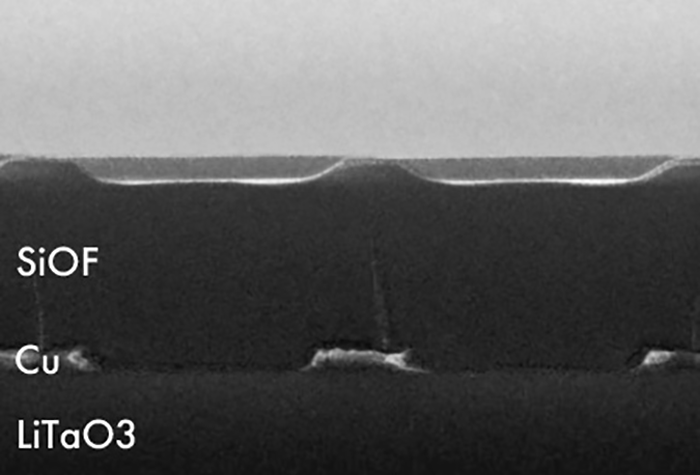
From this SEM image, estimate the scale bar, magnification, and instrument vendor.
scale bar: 1000 nm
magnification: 15 K X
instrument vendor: other